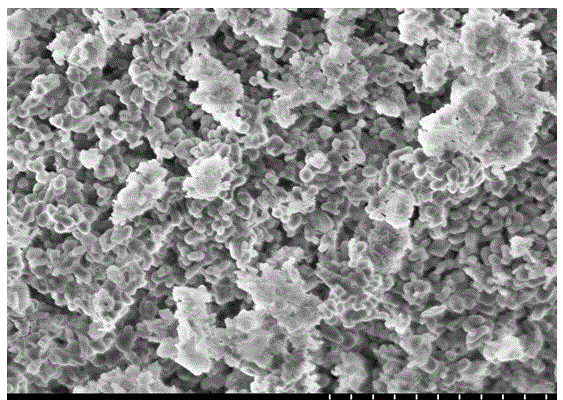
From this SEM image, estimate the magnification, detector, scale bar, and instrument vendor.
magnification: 10 K X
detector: SE(U)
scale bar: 5000 nm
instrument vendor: Hitachi